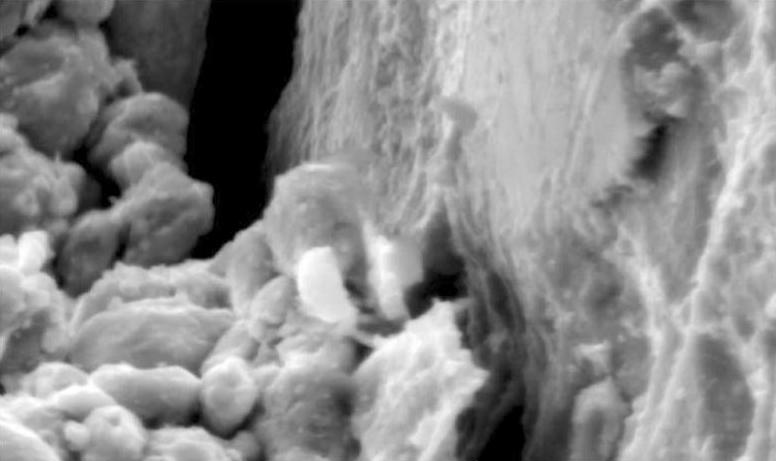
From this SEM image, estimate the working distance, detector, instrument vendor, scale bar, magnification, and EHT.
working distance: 9.7 mm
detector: SE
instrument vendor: FEI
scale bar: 5000 nm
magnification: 10 K X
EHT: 20 kV